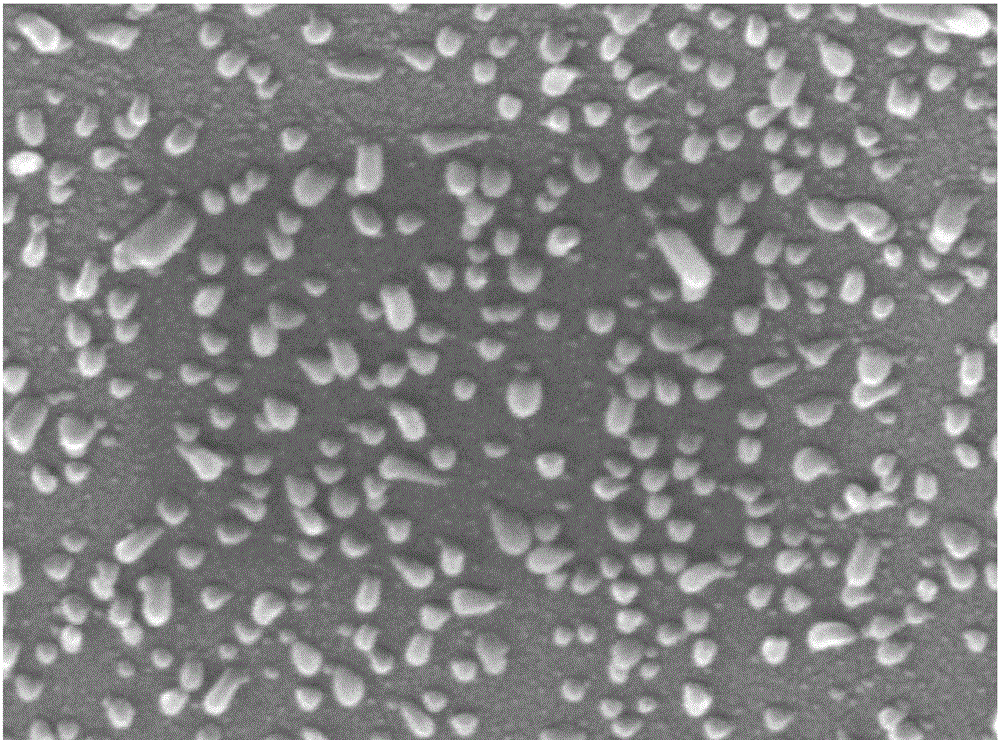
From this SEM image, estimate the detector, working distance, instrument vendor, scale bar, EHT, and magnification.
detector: LED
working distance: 9.9 mm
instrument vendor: JEOL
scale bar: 100 nm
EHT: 15 kV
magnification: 100 K X